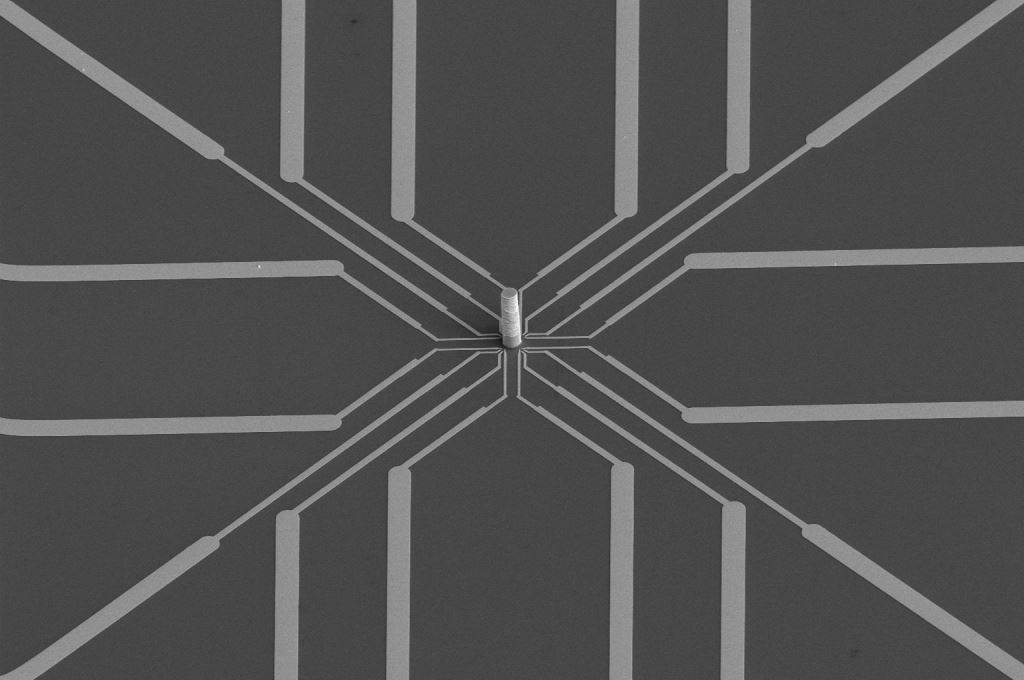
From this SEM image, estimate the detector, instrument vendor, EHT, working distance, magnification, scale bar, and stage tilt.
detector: ETD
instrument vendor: FEI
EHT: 5 kV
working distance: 35.1 mm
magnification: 1.5 K X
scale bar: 40000 nm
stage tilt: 0°